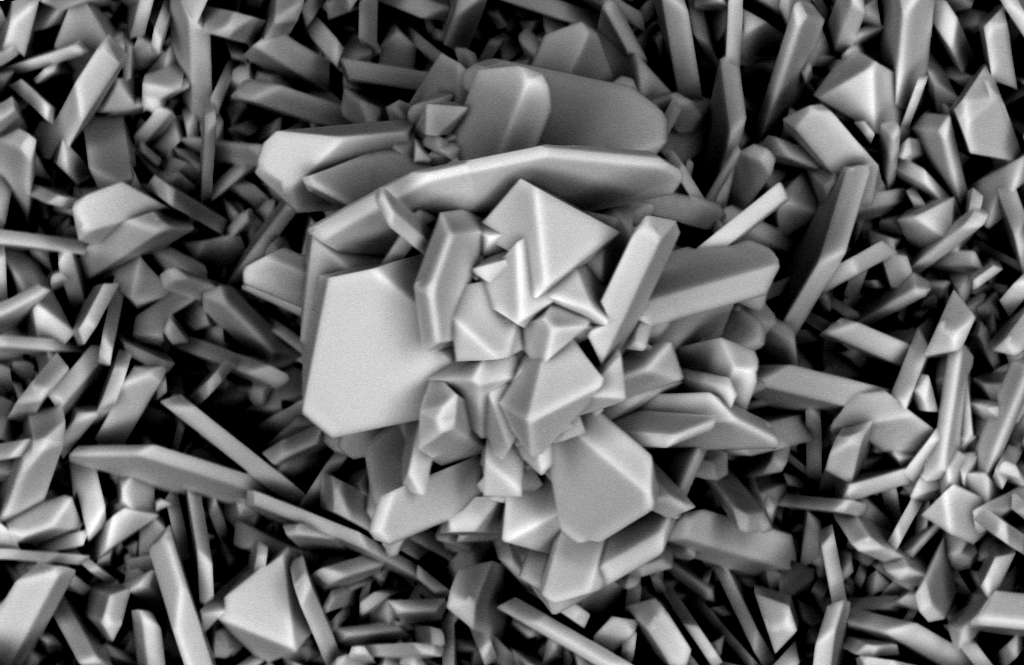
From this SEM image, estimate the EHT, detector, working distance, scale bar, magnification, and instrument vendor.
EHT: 10 kV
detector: BSD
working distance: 7.9 mm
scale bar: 1000 nm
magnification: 3.9 K X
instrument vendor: Zeiss